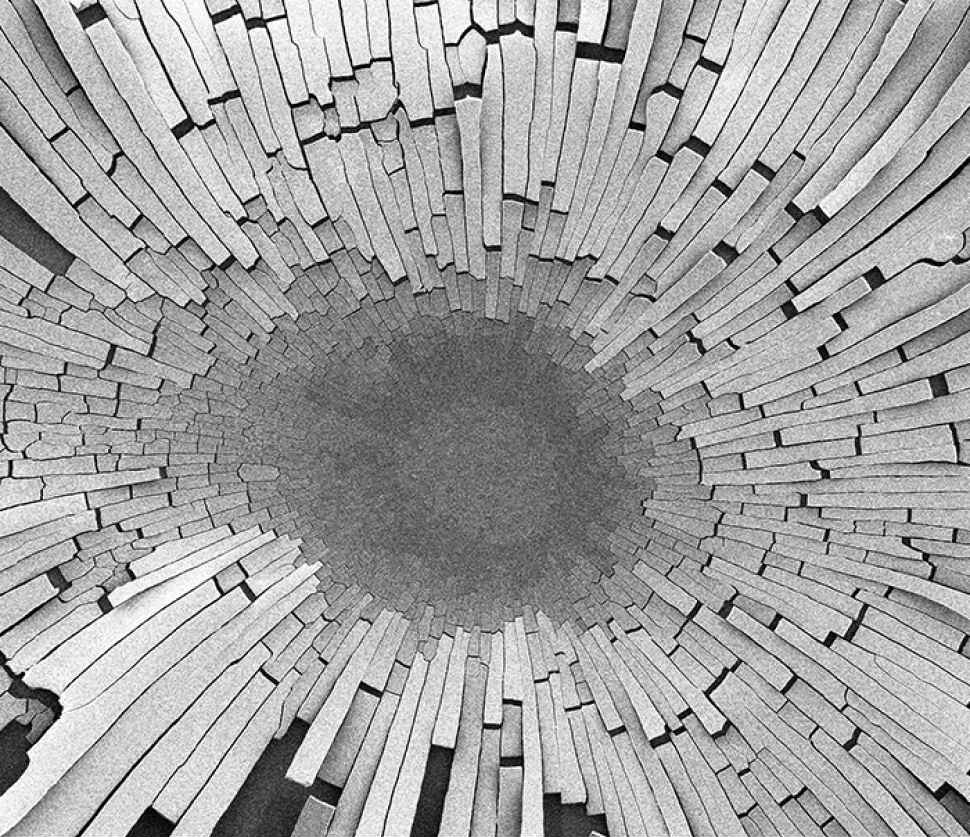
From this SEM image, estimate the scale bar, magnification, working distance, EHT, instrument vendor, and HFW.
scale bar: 300000 nm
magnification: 0.416 K X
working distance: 6.6 mm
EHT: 5 kV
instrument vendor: FEI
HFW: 717 µm